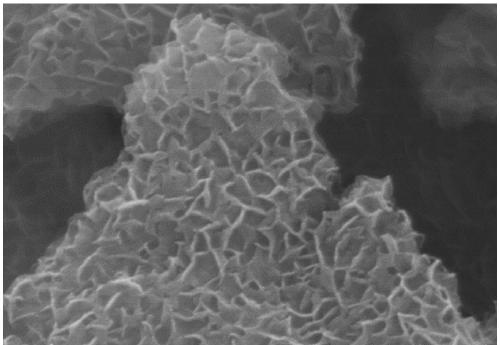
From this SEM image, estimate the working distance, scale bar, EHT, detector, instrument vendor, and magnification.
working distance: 9 mm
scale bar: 500 nm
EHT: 10 kV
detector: SE(M)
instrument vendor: Hitachi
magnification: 100 K X